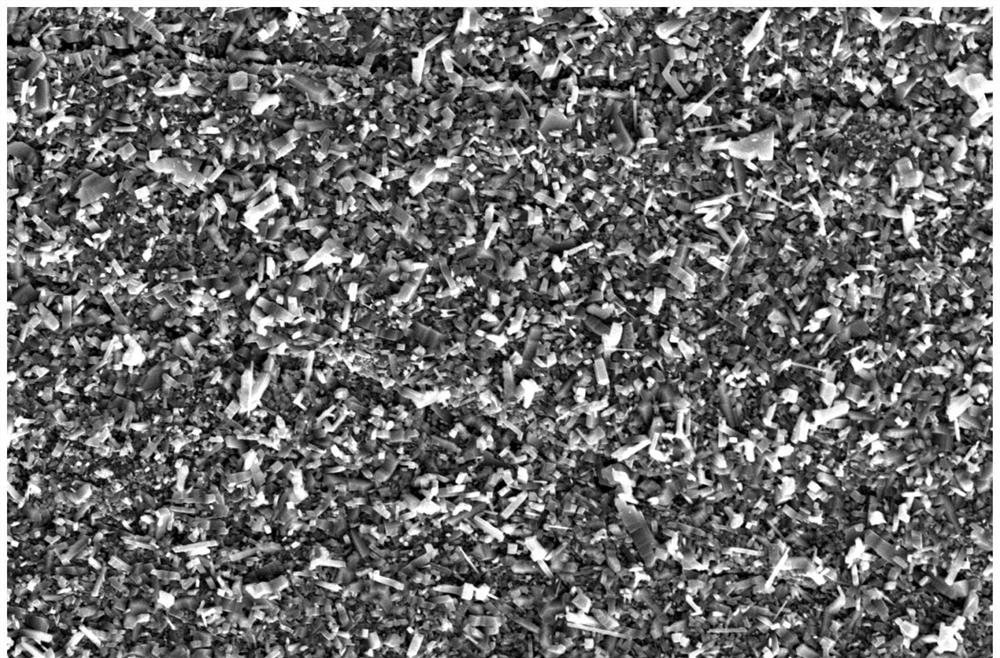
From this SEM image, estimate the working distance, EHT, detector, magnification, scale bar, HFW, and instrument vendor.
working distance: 5.5 mm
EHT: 15 kV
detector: SE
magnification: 3 K X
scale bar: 20000 nm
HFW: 69.1 µm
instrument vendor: FEI